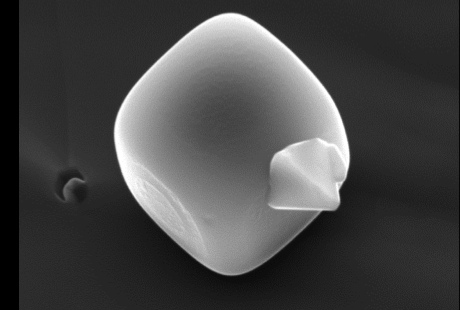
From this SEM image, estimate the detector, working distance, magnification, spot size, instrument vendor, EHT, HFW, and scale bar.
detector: ETD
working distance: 13.5 mm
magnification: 11.613 K X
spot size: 4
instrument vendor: FEI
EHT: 10 kV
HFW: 6.03 µm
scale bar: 1000 nm